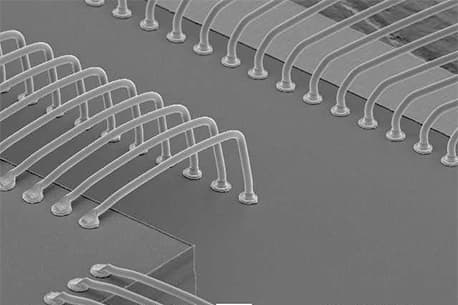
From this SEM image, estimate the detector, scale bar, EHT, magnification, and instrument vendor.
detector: SEI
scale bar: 100000 nm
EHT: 15 kV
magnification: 0.1 K X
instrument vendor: JEOL